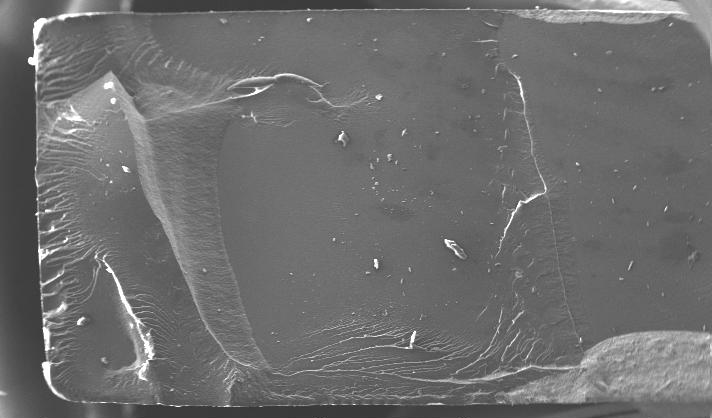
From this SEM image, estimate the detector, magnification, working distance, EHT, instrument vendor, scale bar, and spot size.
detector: SE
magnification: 0.05 K X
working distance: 8.1 mm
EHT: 20 kV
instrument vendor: FEI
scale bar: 1000000 nm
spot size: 6